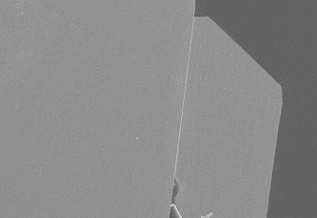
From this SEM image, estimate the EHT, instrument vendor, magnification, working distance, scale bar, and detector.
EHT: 10 kV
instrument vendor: Hitachi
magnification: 0.05 K X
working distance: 15.2 mm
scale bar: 1000000 nm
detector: SE